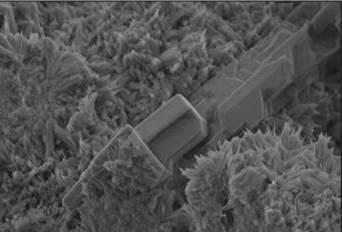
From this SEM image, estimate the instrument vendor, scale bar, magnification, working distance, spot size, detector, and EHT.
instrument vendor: FEI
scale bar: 1000 nm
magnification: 16 K X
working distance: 3.8 mm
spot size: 2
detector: SE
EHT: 2.5 kV